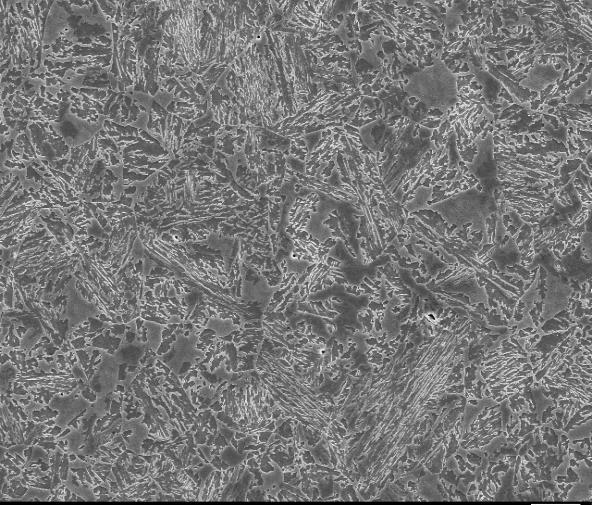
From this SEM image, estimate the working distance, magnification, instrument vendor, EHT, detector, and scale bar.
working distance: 24.6 mm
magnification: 1 K X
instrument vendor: JEOL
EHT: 20 kV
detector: SEI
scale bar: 10000 nm